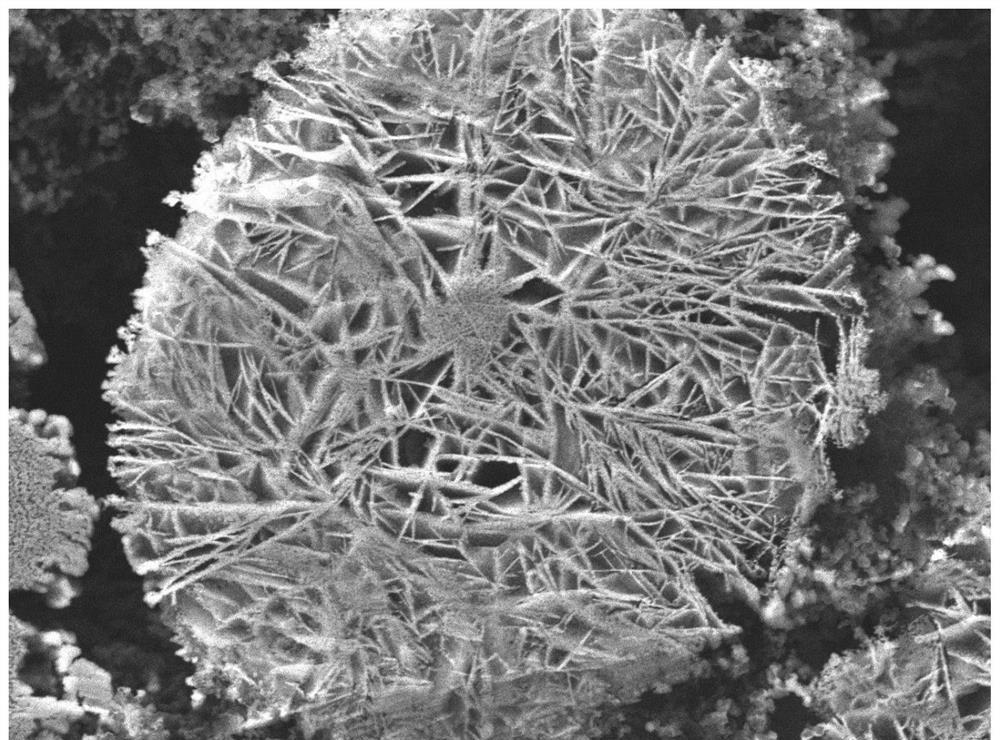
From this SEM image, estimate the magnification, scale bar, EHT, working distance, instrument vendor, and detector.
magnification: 13 K X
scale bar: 1000 nm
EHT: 5 kV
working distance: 9 mm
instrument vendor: JEOL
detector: LEI